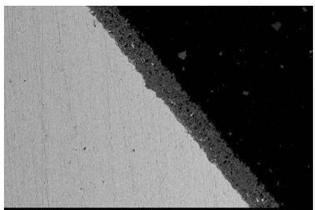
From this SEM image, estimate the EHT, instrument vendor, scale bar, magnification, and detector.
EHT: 20 kV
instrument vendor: JEOL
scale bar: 100000 nm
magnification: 0.1 K X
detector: BEC